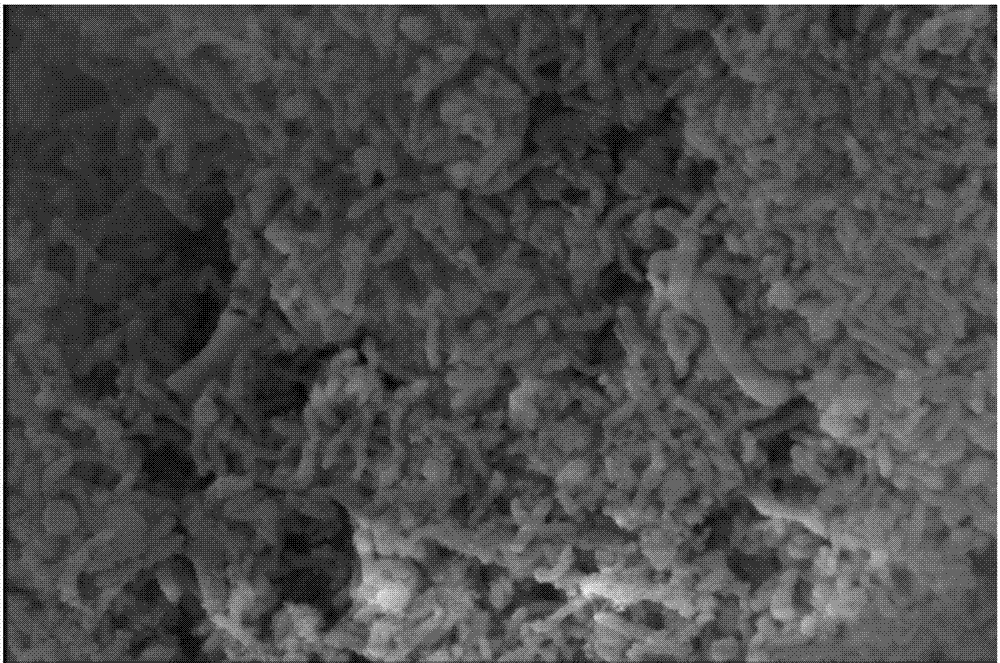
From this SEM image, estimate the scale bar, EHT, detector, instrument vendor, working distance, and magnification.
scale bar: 5000 nm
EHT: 10 kV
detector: ETD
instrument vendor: FEI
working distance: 30 mm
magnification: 10 K X